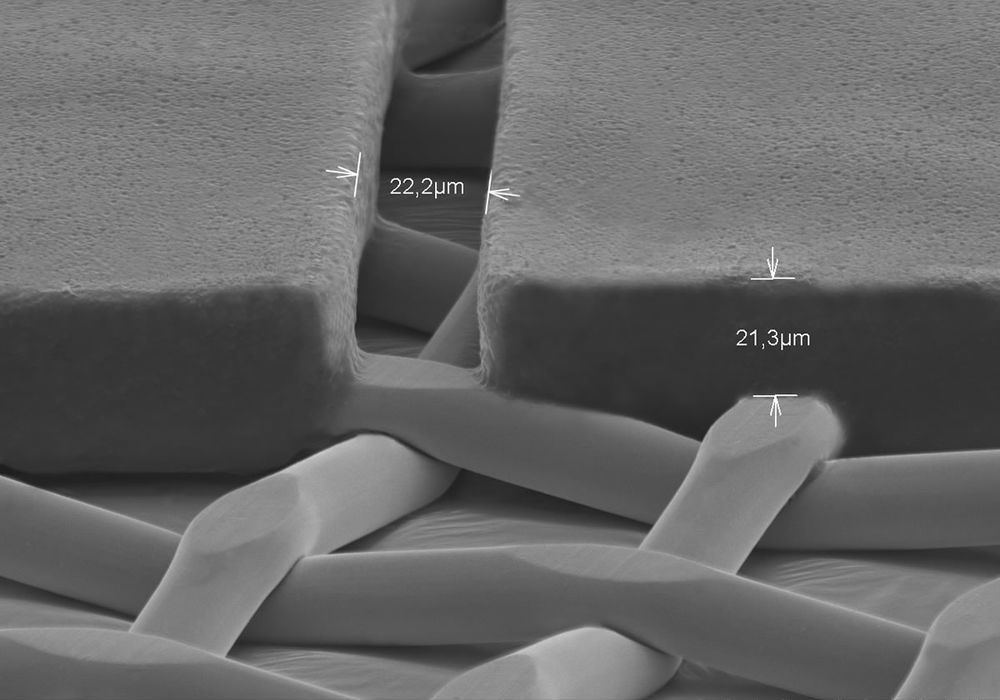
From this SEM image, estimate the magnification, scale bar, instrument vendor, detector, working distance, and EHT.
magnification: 0.7 K X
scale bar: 50000 nm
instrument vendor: Hitachi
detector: SE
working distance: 32.9 mm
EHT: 30 kV